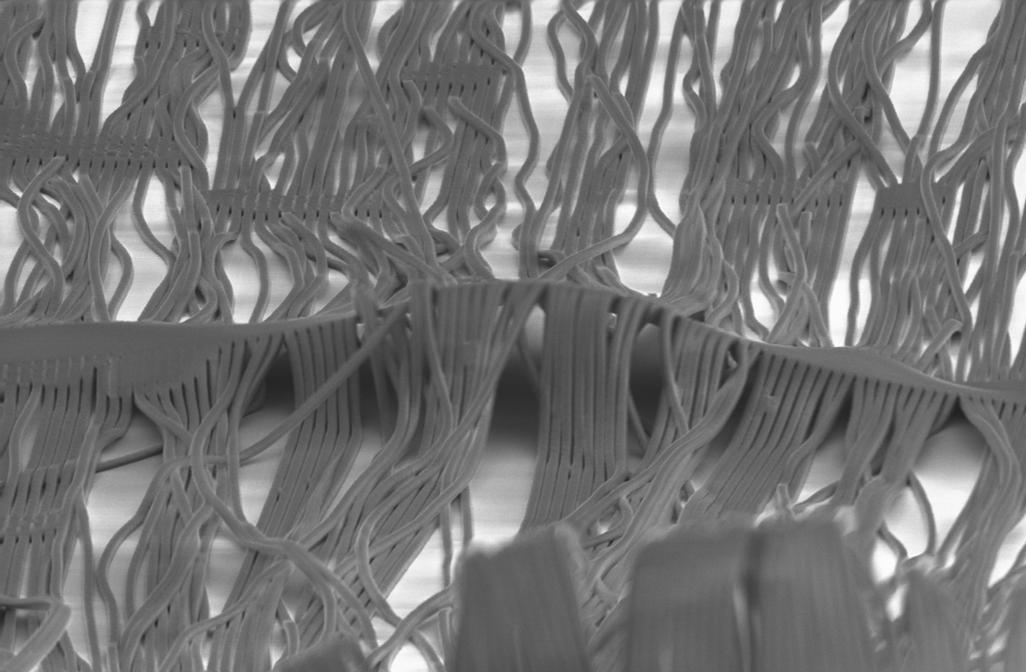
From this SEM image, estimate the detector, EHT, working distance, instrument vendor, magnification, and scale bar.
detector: SE2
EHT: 5 kV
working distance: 11 mm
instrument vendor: Zeiss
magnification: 5.54 K X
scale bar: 10000 nm